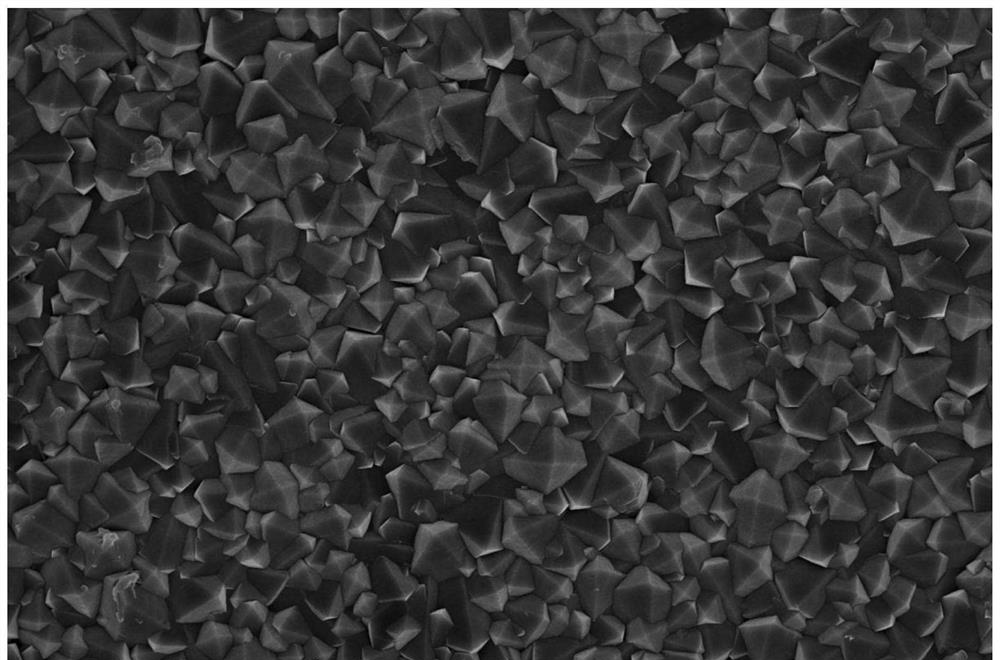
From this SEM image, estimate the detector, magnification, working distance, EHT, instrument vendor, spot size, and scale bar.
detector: TLD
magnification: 10 K X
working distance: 5.2 mm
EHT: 10 kV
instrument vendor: FEI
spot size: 3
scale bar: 10000 nm